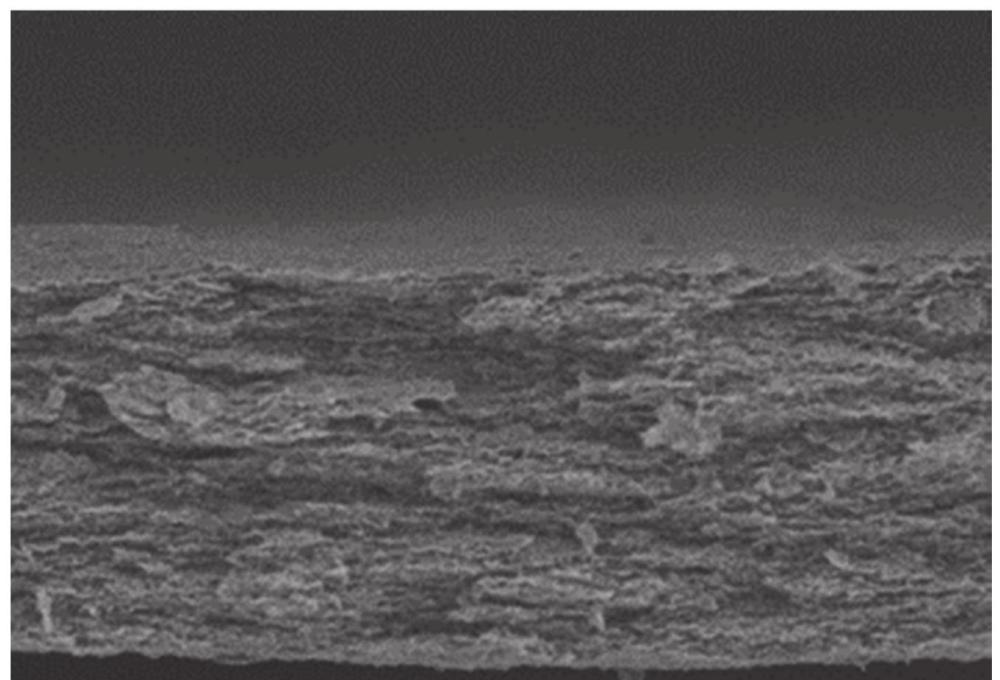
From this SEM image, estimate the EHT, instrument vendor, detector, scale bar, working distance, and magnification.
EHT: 5 kV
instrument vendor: Hitachi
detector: SE(UL)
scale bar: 30000 nm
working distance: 10.2 mm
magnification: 1.5 K X